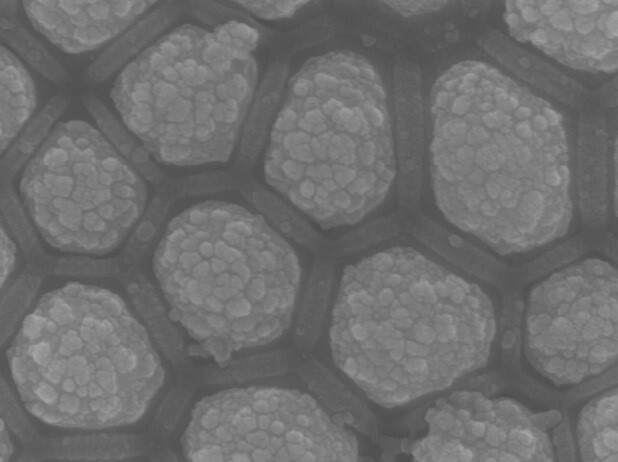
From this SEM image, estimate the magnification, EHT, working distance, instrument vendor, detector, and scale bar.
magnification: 80 K X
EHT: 15 kV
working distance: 8 mm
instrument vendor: JEOL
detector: SEI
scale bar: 100 nm